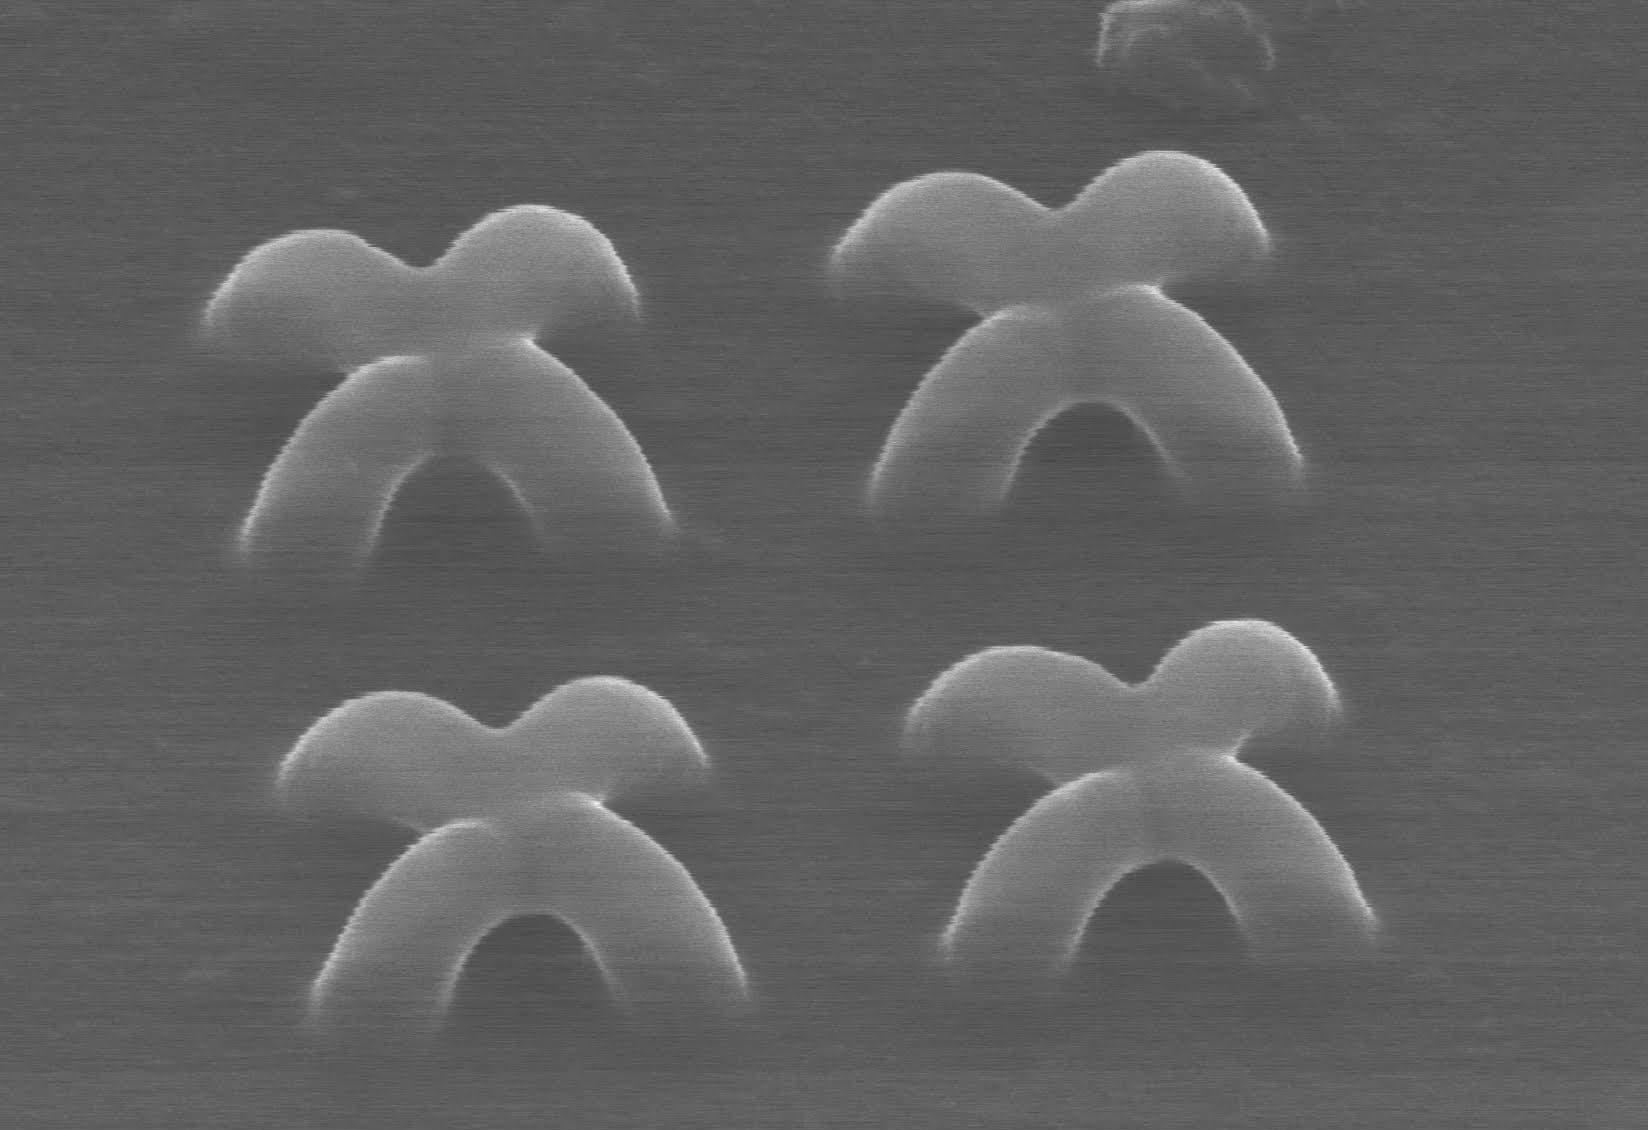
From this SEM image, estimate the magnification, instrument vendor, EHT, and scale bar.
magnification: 40 K X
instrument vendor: Hitachi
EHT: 20 kV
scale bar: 750 nm